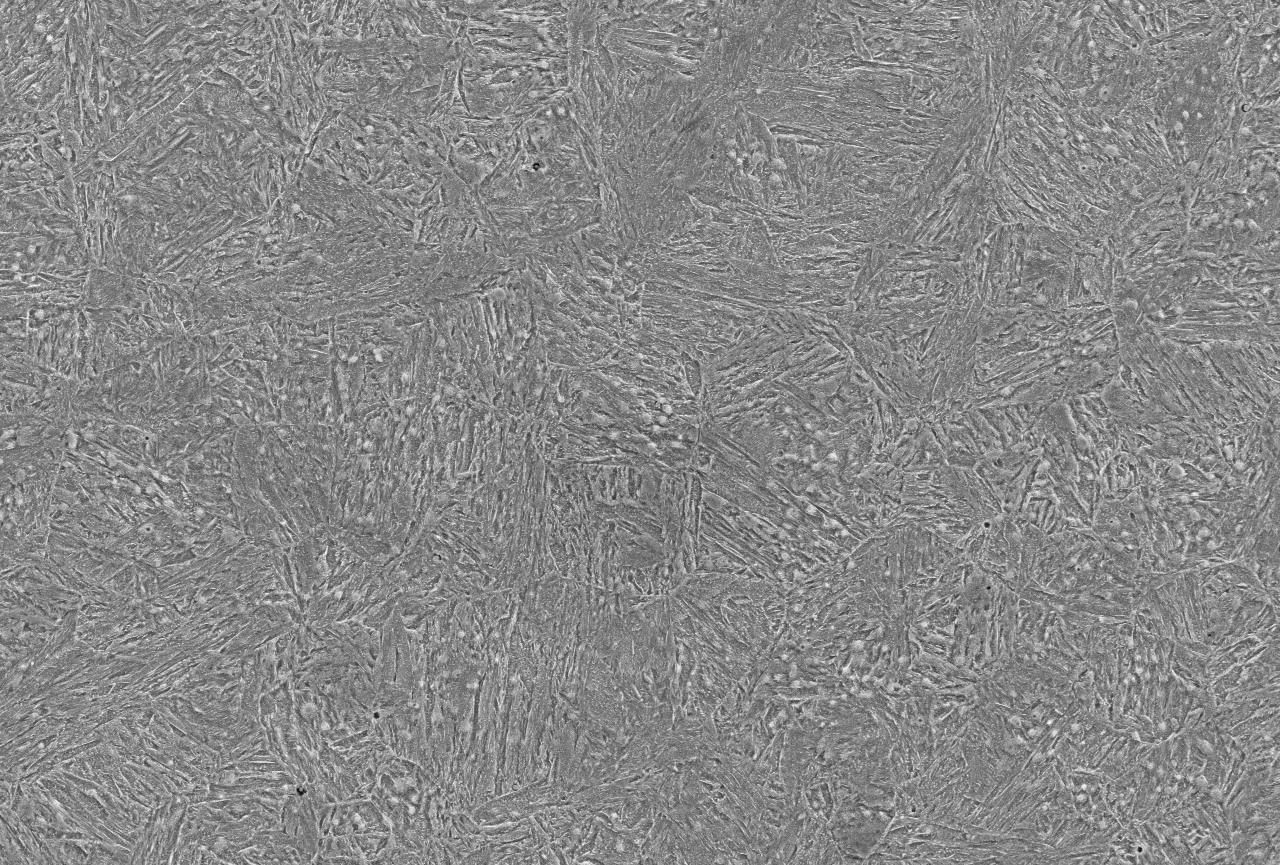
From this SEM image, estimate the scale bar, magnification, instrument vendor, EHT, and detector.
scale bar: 20000 nm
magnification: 1 K X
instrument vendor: JEOL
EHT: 15 kV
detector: SEI